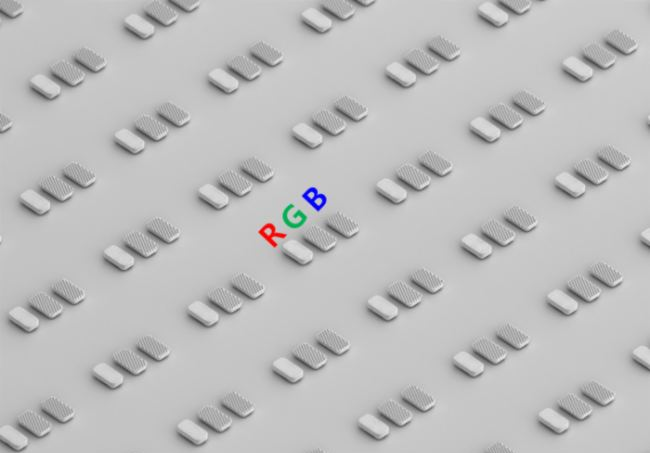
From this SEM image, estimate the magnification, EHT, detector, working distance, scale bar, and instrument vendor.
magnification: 0.2 K X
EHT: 15 kV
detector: UD(+LD)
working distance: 19.6 mm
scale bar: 200000 nm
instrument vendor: Hitachi